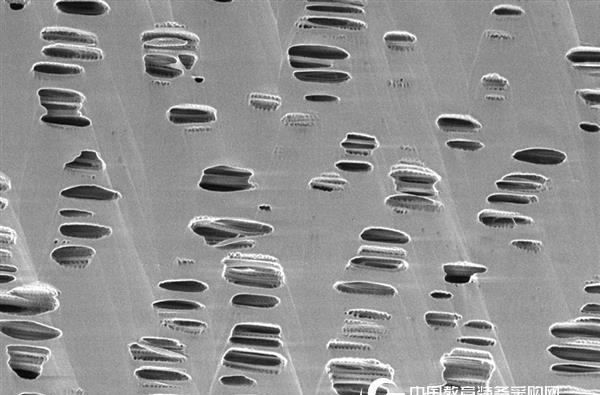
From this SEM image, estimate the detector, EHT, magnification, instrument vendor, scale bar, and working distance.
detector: InLens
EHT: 0.5 kV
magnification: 20 K X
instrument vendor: Zeiss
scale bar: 200 nm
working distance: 1.7 mm